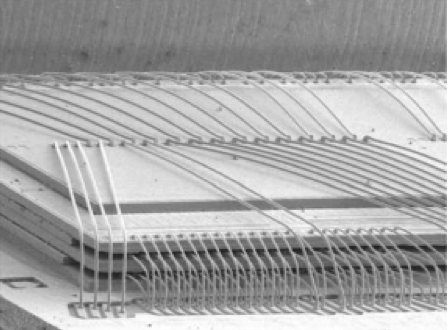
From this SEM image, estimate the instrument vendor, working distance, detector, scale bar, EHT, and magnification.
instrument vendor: JEOL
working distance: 27 mm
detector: LEI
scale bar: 1000000 nm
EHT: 15 kV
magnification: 0.025 K X